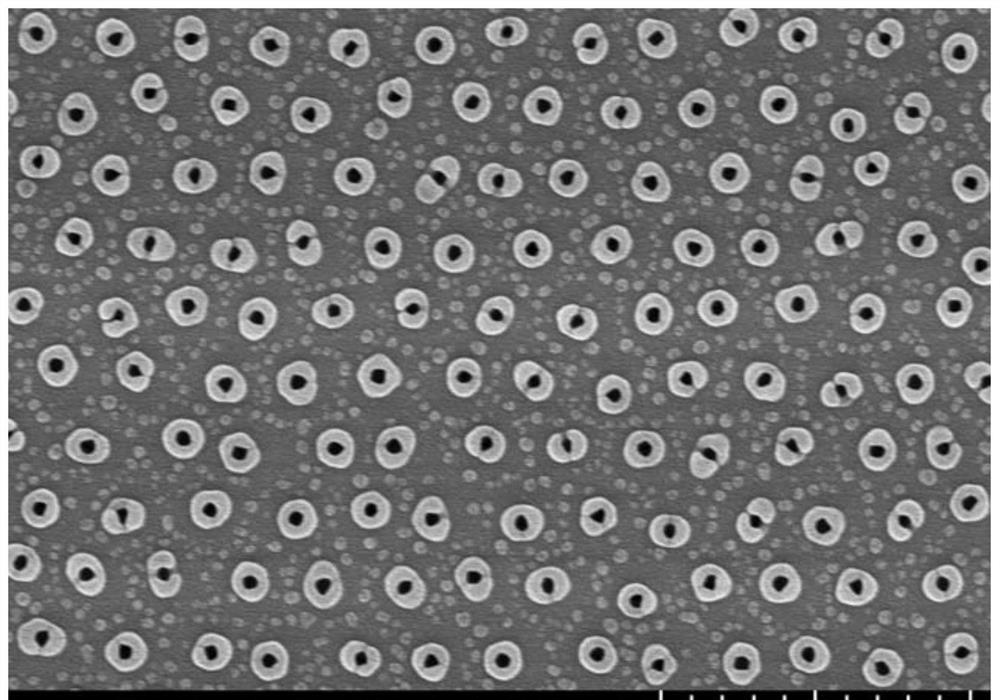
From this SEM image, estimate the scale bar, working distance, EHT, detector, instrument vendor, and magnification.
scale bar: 2000 nm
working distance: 9.3 mm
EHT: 10 kV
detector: SE(UL)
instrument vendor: Hitachi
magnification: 20 K X